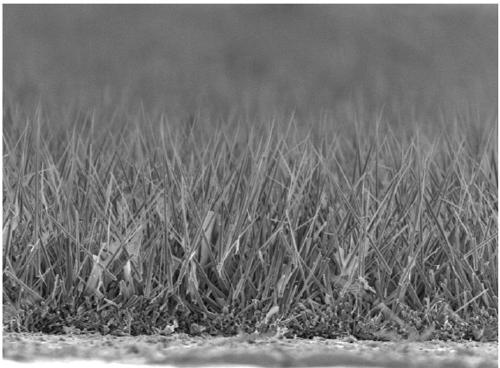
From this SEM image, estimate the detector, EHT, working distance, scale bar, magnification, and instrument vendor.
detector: LED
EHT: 3 kV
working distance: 8 mm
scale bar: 1000 nm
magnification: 4 K X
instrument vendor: JEOL